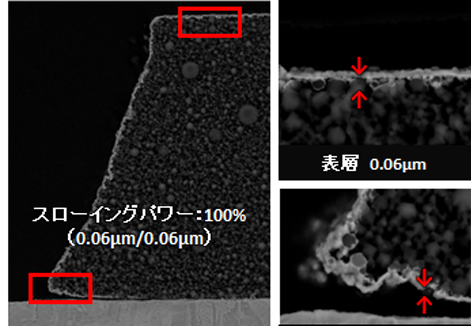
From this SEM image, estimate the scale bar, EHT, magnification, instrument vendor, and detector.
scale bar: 1000 nm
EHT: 5 kV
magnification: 5 K X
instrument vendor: JEOL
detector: BED-C